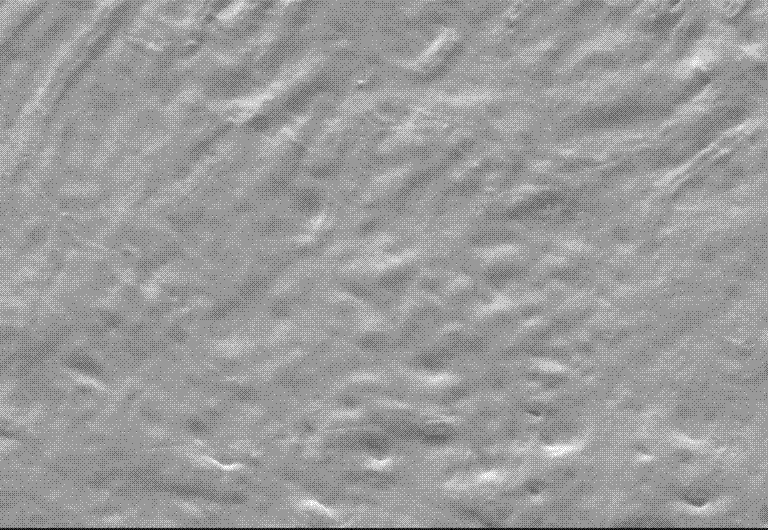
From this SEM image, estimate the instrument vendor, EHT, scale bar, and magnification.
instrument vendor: other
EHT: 25 kV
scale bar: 10000 nm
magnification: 2 K X